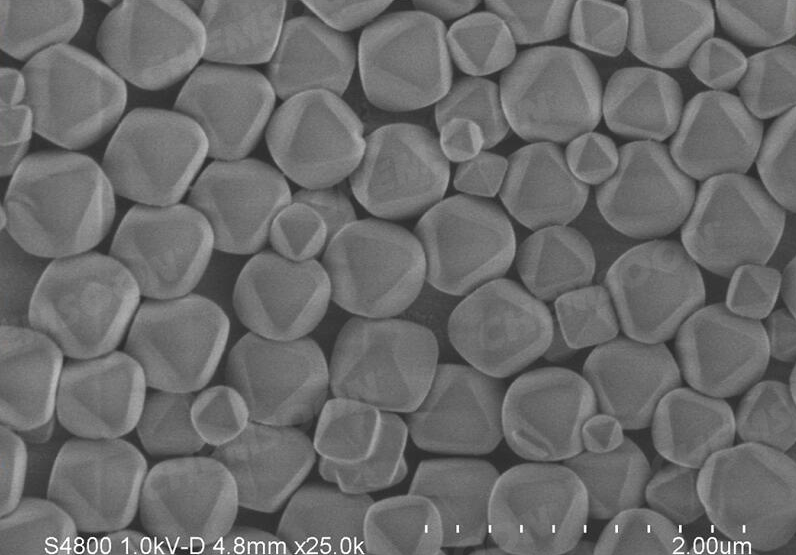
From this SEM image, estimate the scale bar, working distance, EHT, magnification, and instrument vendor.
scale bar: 2000 nm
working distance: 4.8 mm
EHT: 1 kV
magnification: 25 K X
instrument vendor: Hitachi